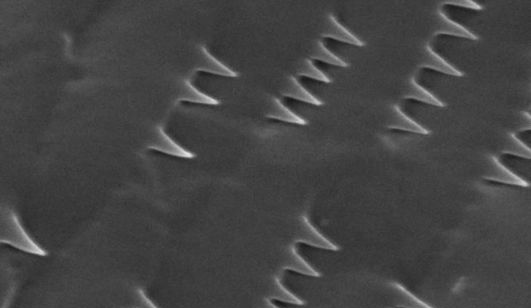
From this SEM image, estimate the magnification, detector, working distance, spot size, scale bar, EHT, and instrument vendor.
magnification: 4.788 K X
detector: SE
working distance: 32.7 mm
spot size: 3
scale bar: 5000 nm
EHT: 25 kV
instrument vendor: FEI